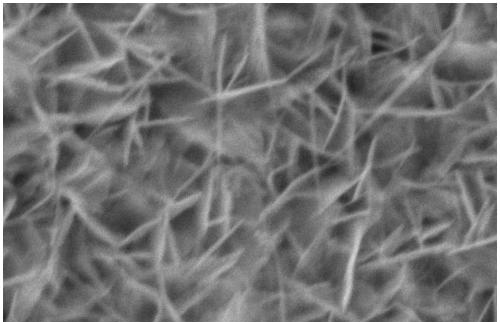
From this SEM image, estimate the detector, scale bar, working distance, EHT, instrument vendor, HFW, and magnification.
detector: ETD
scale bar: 500 nm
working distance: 11.4 mm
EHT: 10 kV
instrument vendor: FEI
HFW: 2.54 µm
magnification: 50 K X